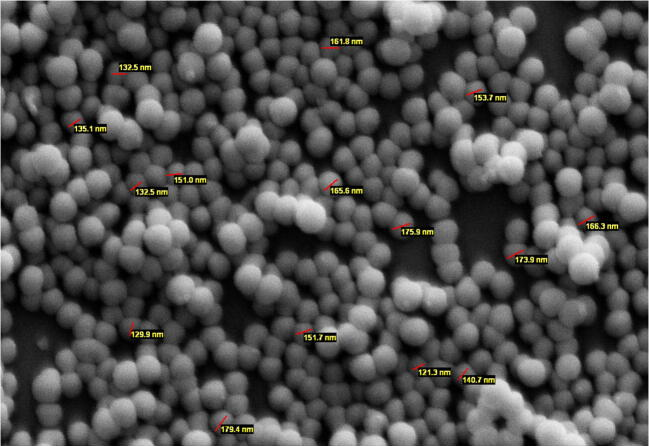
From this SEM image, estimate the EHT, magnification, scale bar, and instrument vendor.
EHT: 25 kV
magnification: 20 K X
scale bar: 1000 nm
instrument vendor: other